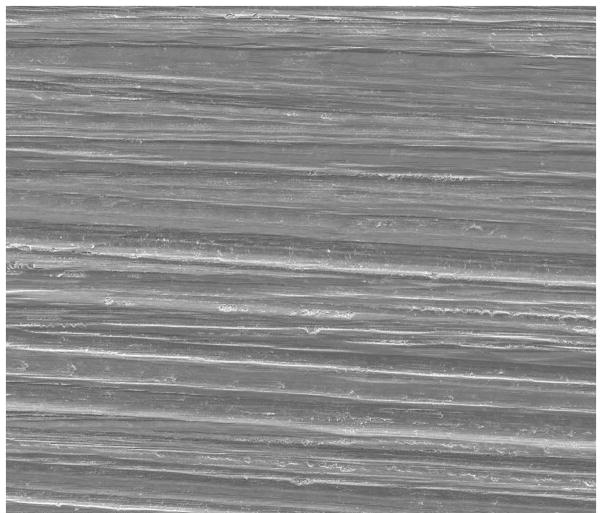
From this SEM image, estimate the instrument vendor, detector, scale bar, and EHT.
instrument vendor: FEI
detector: ETD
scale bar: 400000 nm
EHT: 20 kV